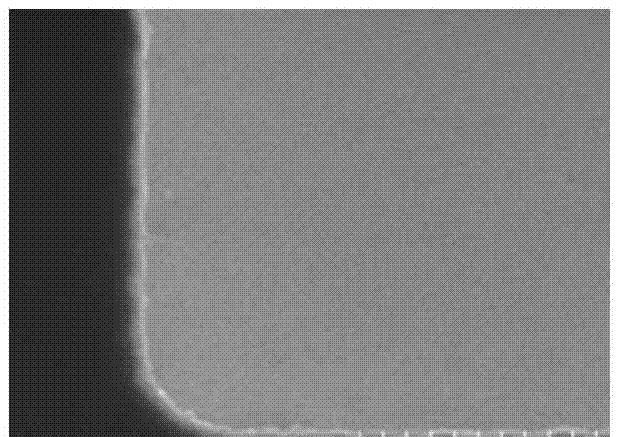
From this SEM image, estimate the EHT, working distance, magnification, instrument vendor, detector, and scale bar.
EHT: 15 kV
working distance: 9.3 mm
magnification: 100 K X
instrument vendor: Hitachi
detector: SE(U)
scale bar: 500 nm